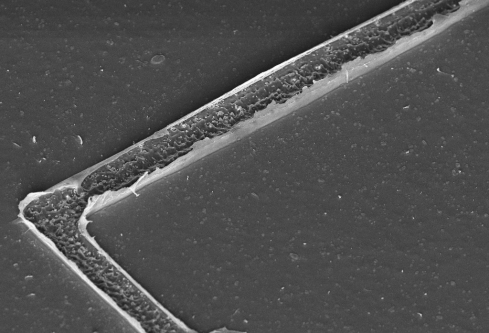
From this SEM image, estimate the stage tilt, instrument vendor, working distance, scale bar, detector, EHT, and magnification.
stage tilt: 45°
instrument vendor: Zeiss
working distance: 8.7 mm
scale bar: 10000 nm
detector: InLens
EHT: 5 kV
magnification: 2.56 K X